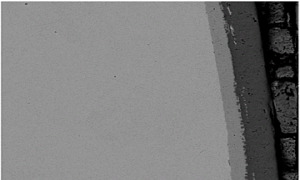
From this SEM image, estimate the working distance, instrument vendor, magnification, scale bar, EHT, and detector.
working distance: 11 mm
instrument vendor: JEOL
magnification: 1 K X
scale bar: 10000 nm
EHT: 15 kV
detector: COMPO